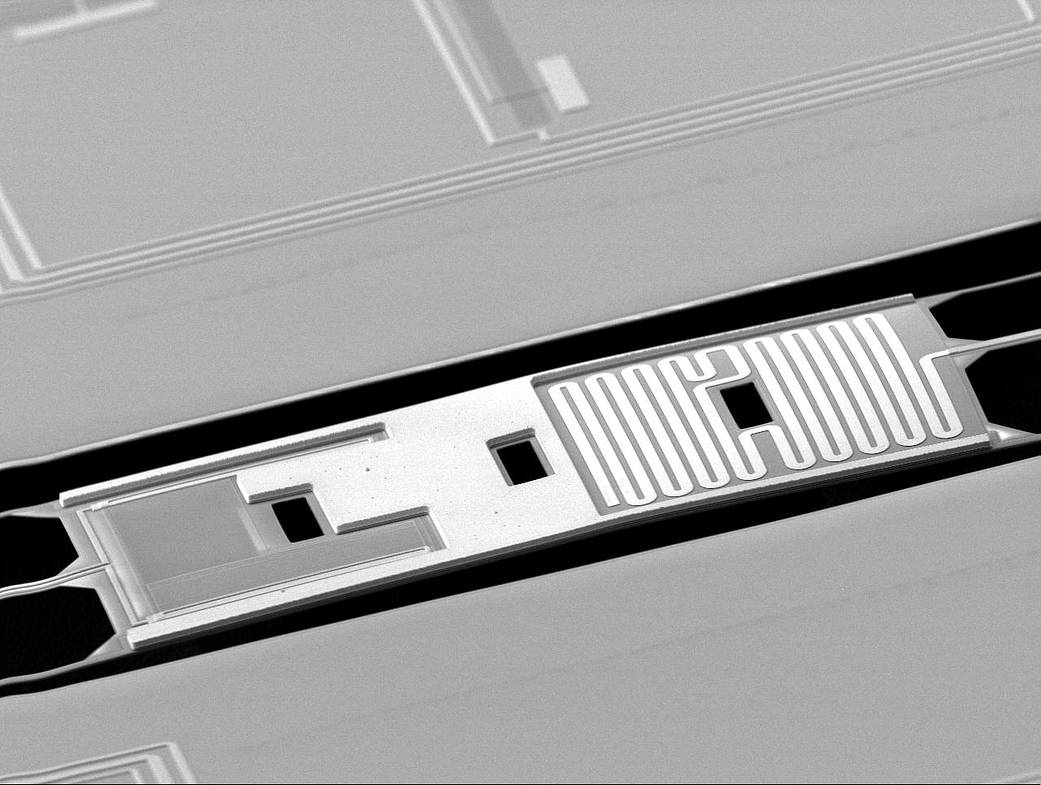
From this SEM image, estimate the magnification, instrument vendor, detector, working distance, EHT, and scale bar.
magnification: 0.33 K X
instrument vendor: JEOL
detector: LEI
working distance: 17.8 mm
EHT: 10 kV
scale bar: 10000 nm